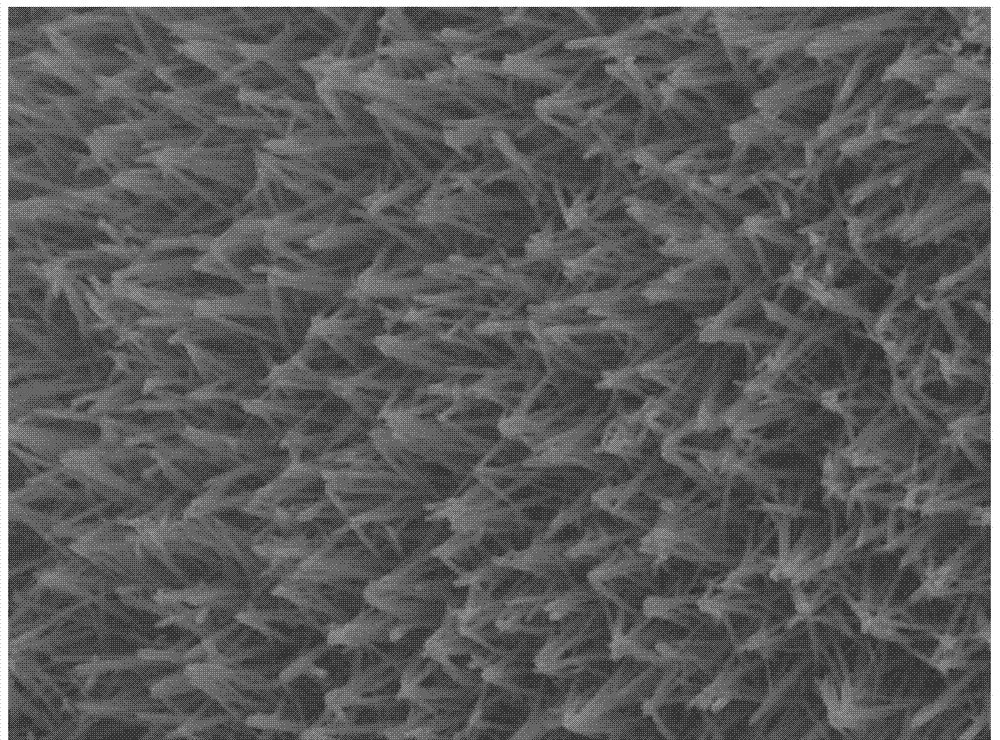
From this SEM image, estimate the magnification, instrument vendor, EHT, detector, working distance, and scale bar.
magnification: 20 K X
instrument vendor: JEOL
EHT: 15 kV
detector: SEI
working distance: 10.2 mm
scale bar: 1000 nm